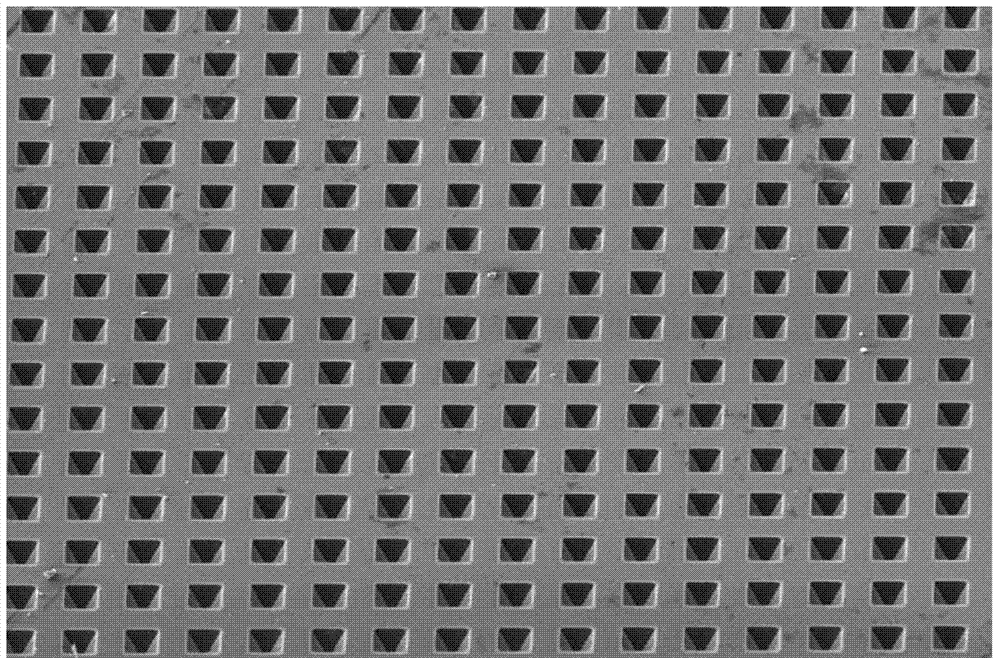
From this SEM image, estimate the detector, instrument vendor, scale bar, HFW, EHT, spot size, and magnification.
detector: ETD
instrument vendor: FEI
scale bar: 100000 nm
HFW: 319 µm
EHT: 10 kV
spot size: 3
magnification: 1.3 K X